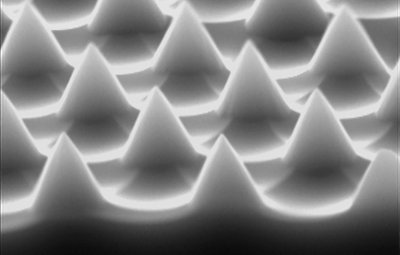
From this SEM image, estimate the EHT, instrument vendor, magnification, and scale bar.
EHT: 15 kV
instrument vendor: JEOL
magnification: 10 K X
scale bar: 1000 nm